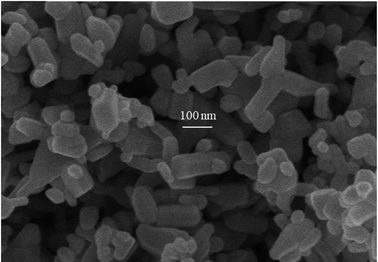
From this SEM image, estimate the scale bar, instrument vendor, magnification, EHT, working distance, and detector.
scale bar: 500 nm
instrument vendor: Hitachi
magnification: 100 K X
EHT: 5 kV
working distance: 4.9 mm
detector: SE(M)